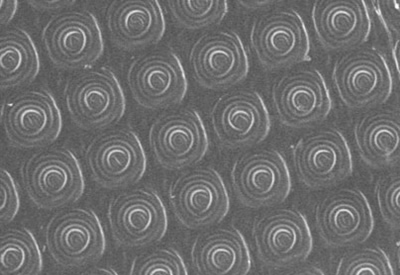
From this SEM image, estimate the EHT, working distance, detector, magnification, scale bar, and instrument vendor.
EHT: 3 kV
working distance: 15 mm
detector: SEI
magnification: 3 K X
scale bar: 1000 nm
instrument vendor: JEOL